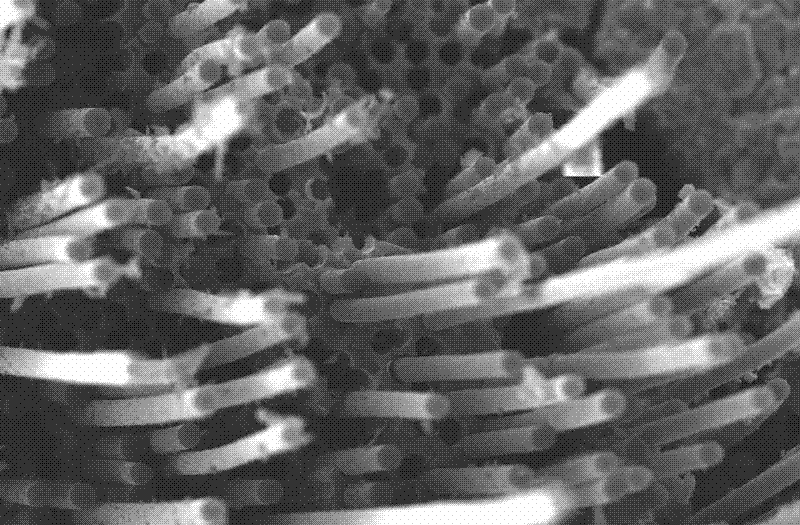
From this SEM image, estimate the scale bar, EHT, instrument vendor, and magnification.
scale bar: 20000 nm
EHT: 20 kV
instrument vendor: JEOL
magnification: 0.6 K X